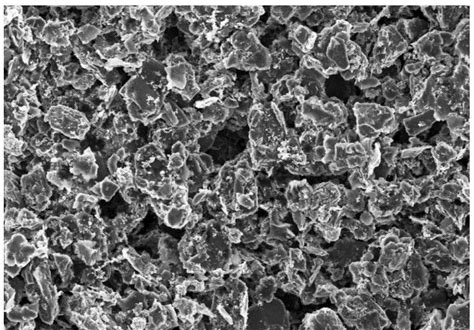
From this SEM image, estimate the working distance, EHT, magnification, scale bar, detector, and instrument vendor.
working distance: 7.7 mm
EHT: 5 kV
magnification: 2 K X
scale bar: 20000 nm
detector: SE(M)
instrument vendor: Hitachi